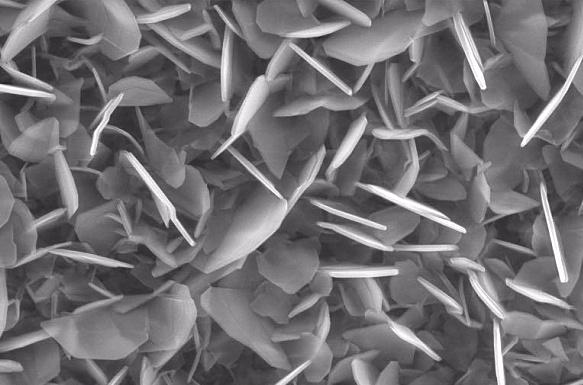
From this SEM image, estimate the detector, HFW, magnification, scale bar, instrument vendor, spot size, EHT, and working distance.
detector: ETD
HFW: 8.29 µm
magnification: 50 K X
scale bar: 2000 nm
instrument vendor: FEI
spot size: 4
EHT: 20 kV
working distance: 9.8 mm